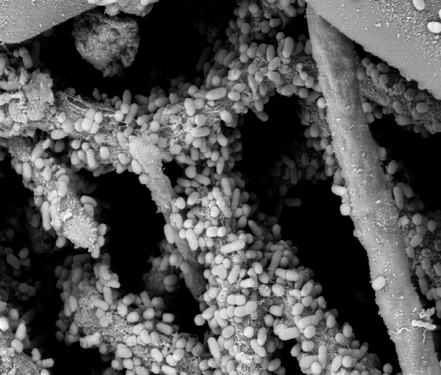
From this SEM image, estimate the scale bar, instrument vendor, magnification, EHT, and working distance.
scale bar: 5000 nm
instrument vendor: JEOL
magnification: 5 K X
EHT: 8 kV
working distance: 14 mm